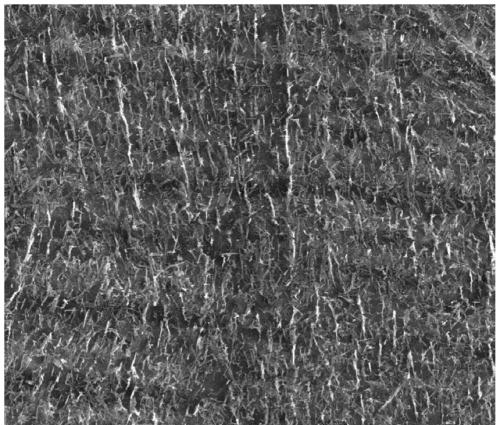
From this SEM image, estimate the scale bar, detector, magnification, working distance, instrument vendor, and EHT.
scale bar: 5000 nm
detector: SE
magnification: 13 K X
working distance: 6.5 mm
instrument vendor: FEI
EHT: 20 kV